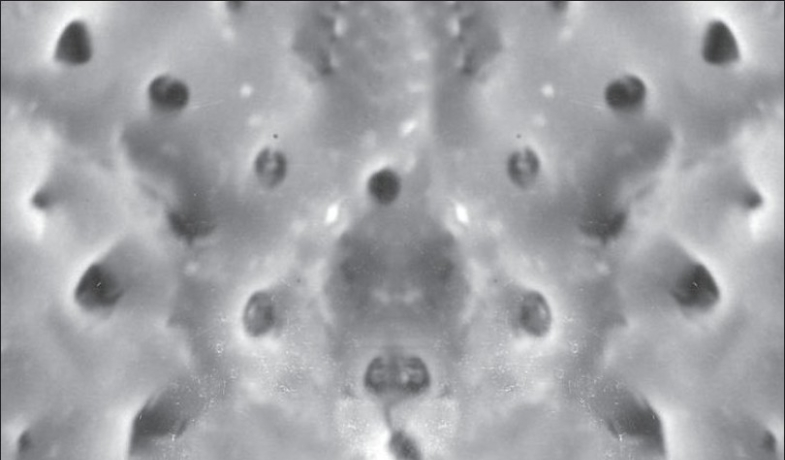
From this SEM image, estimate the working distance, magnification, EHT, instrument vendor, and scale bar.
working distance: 18 mm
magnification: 3.5 K X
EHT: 20 kV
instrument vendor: JEOL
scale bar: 10000 nm